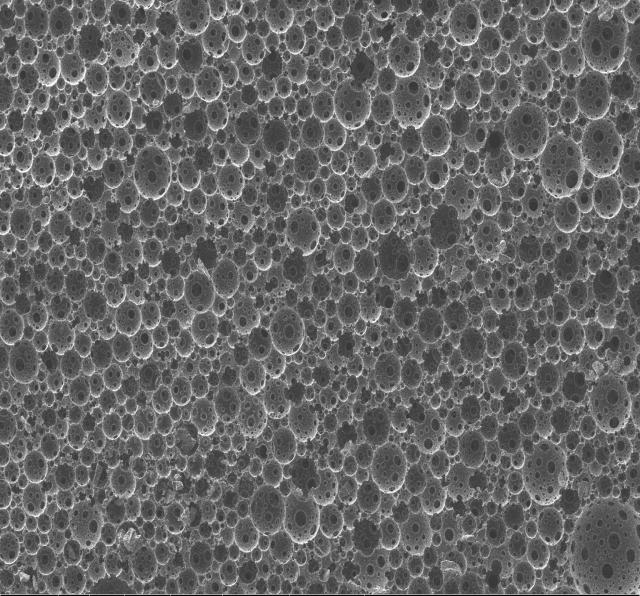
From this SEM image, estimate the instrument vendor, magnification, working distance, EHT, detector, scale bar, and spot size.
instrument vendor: FEI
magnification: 0.3 K X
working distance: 9.9 mm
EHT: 10 kV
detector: ETD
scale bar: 500000 nm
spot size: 3